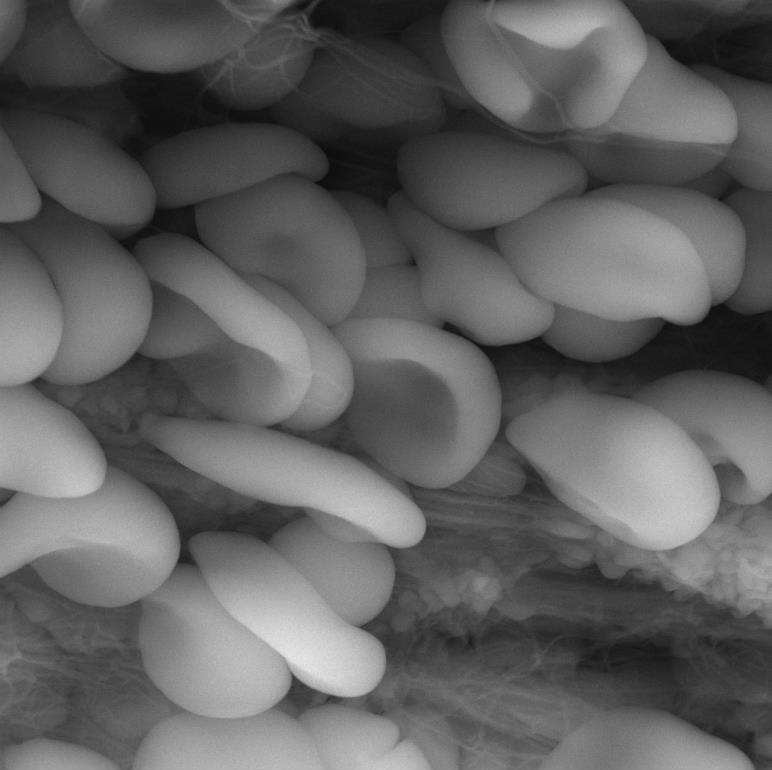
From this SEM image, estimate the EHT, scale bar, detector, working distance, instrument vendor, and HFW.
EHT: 20 kV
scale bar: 5000 nm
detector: LVSTD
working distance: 4.96 mm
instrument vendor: Tescan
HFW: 20 µm